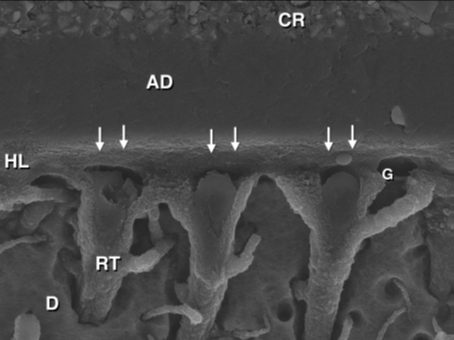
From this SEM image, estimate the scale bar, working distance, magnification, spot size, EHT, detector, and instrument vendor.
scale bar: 10000 nm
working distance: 9.9 mm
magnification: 10 K X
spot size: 2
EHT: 10 kV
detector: ETD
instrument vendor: FEI